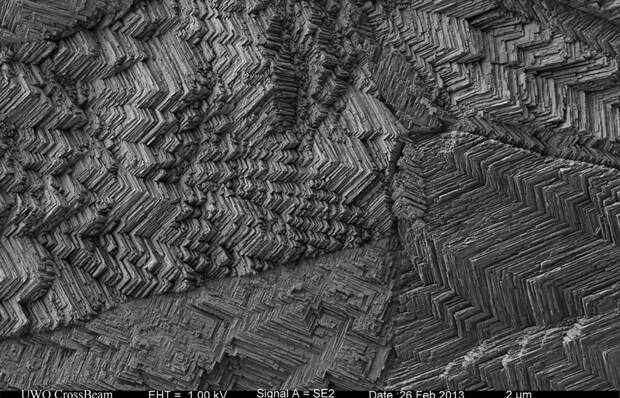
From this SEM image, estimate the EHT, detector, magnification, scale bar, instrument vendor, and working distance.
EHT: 1 kV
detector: SE2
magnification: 2.5 K X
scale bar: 2000 nm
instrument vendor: Zeiss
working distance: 4.8 mm